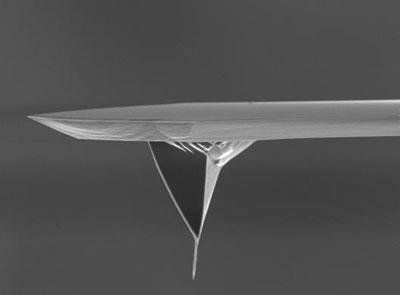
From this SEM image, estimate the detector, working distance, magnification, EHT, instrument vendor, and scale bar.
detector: SEI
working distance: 11.5 mm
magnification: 2.3 K X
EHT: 5 kV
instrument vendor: JEOL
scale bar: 10000 nm